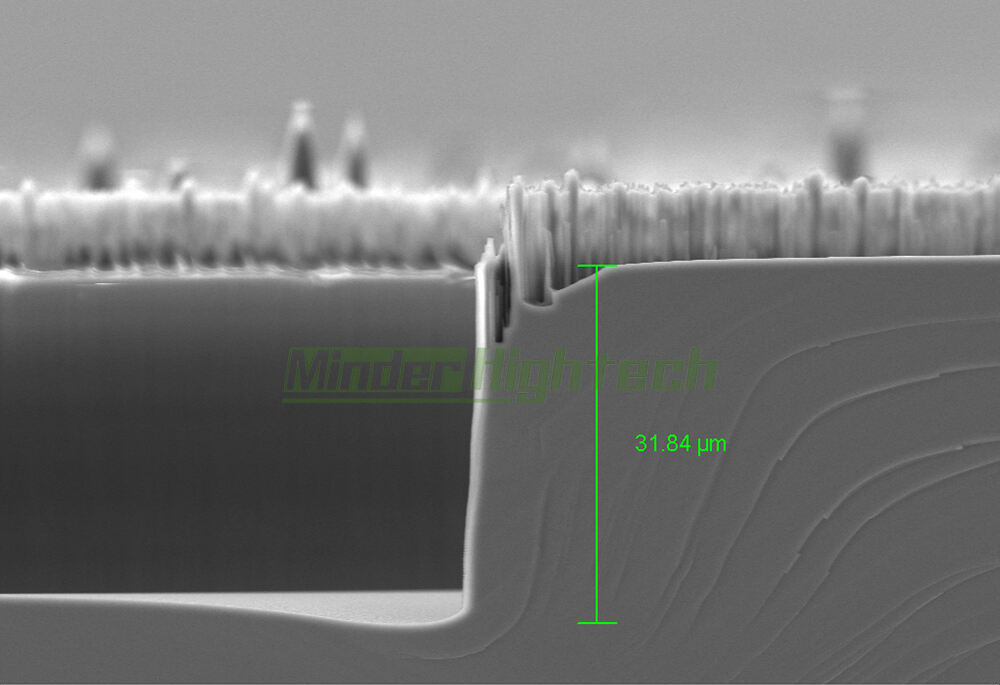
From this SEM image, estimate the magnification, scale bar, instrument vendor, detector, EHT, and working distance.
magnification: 1.28 K X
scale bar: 10000 nm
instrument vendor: Zeiss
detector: SE2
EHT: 10 kV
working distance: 9.1 mm